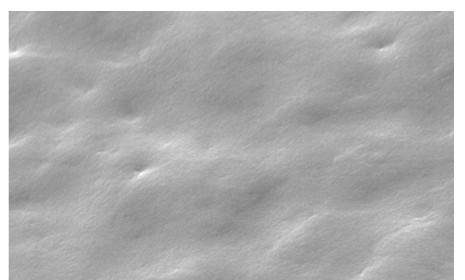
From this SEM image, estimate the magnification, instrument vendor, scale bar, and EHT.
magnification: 2 K X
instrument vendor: other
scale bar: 10000 nm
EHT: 20 kV